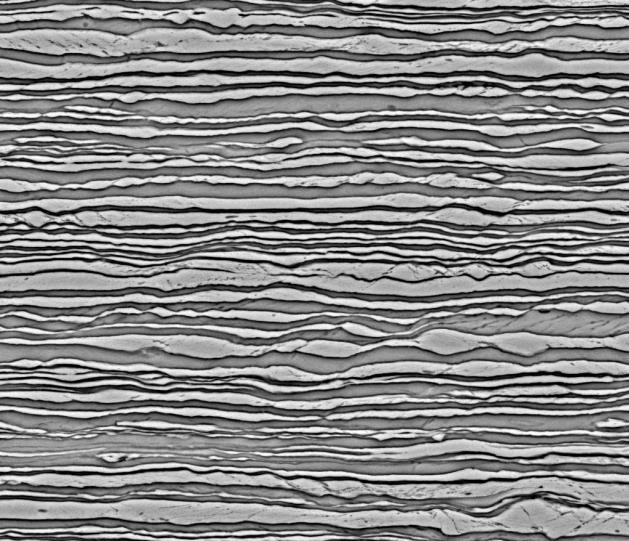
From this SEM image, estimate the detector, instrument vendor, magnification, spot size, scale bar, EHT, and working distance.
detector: BSED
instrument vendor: FEI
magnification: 5 K X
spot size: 3.5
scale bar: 30000 nm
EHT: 20 kV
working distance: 9.7 mm